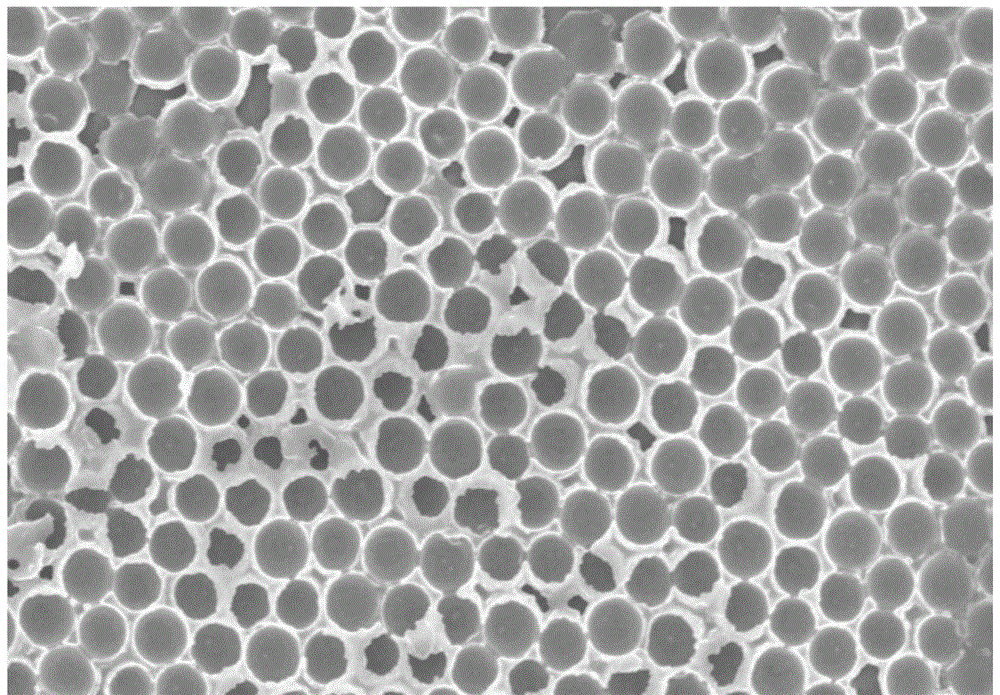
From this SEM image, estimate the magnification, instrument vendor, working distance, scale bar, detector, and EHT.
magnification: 10 K X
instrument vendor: Hitachi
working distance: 12.6 mm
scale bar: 5000 nm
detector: SE(M)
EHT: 15 kV